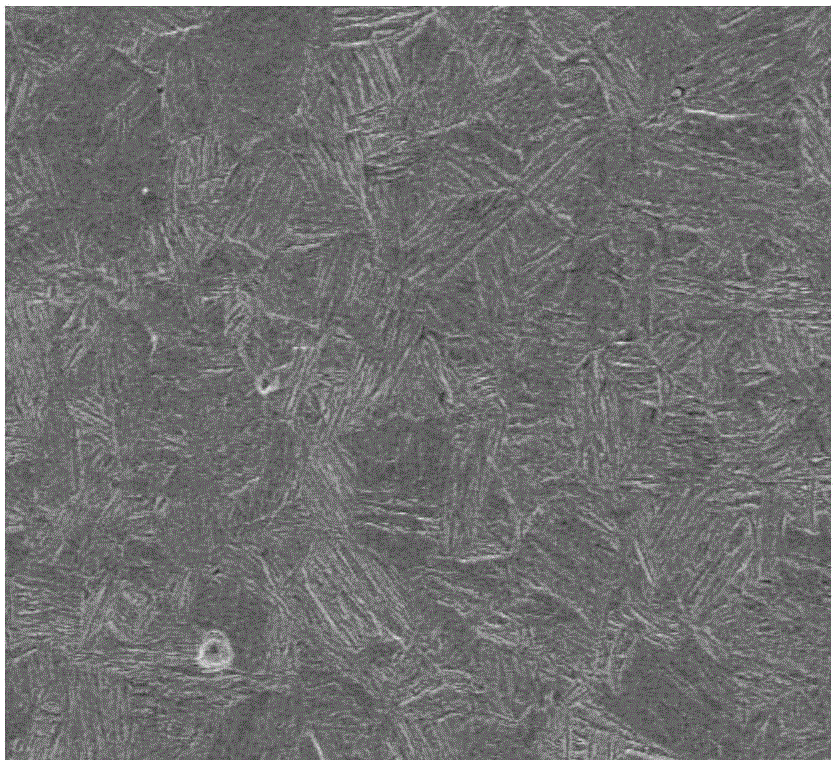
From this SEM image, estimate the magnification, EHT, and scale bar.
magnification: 0.5 K X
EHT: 20 kV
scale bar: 40000 nm